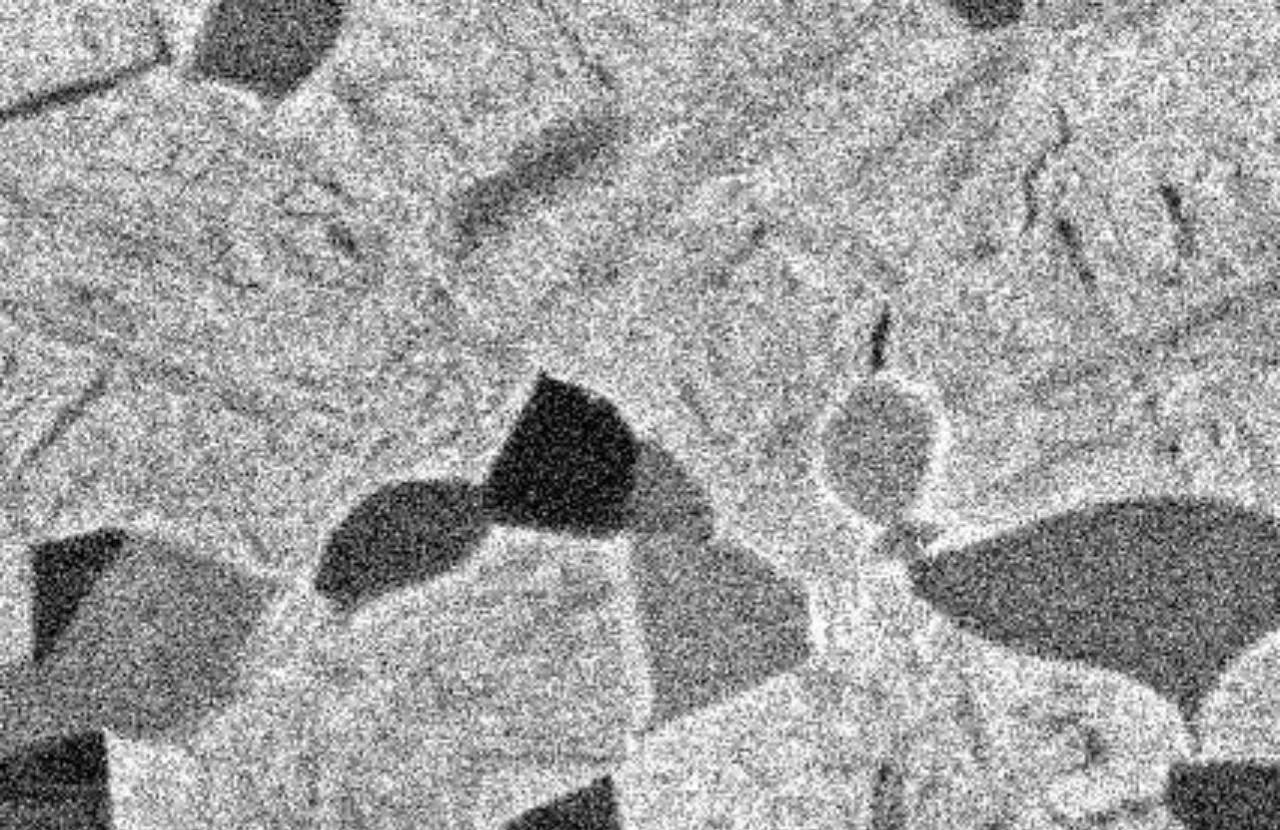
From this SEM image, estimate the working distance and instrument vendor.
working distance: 10 mm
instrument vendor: JEOL